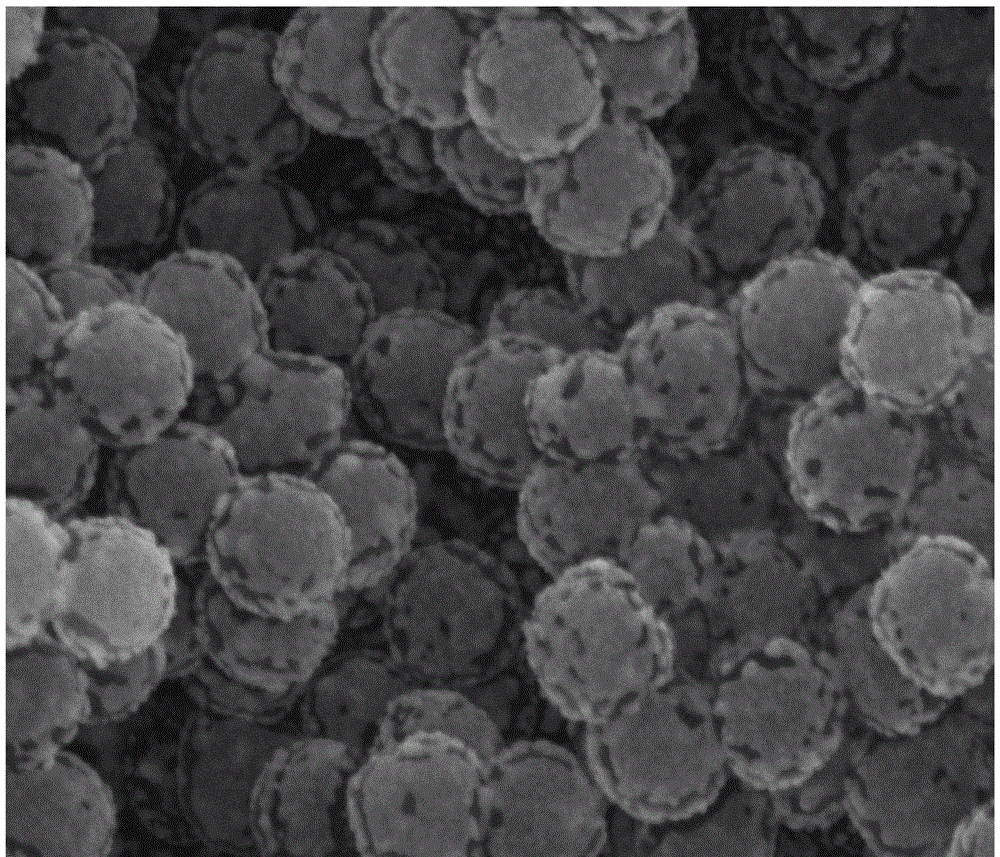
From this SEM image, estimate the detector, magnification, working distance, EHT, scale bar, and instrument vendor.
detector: SE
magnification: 100 K X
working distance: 9.4 mm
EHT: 30 kV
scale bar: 1000 nm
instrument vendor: FEI